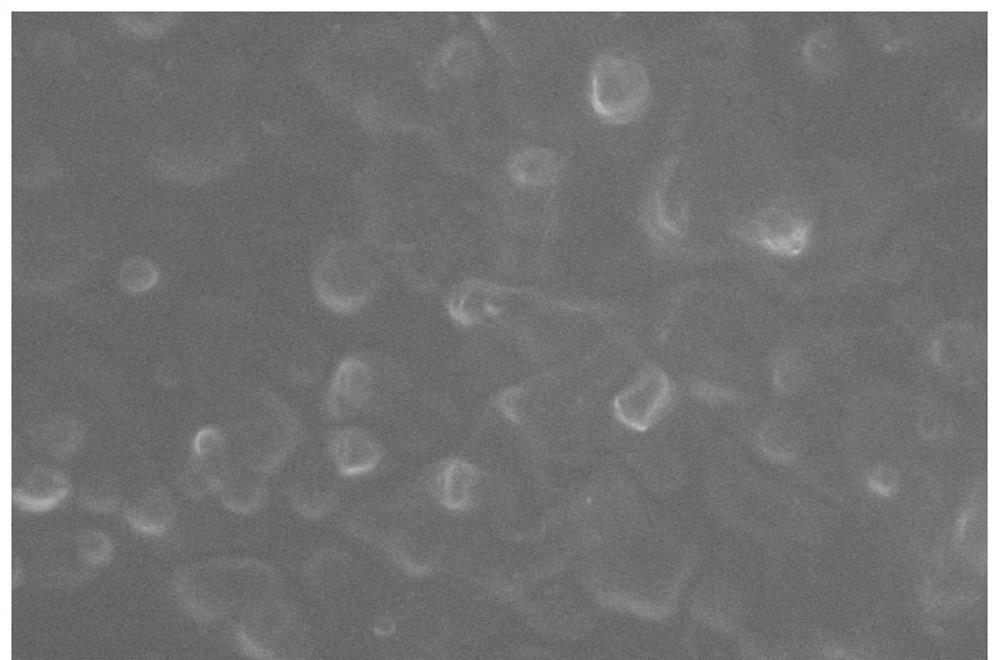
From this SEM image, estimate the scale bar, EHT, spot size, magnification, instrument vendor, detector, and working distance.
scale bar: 3000 nm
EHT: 20 kV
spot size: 3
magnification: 50 K X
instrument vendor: FEI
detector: SE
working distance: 6.6 mm